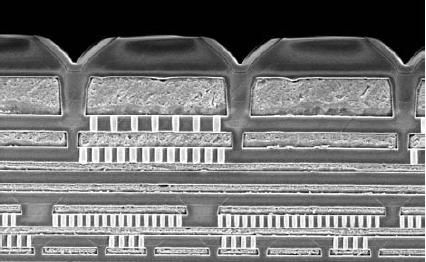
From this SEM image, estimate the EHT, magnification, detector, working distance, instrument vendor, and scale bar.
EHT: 5 kV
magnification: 5 K X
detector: SE(U)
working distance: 5.7 mm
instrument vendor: Hitachi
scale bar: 10000 nm